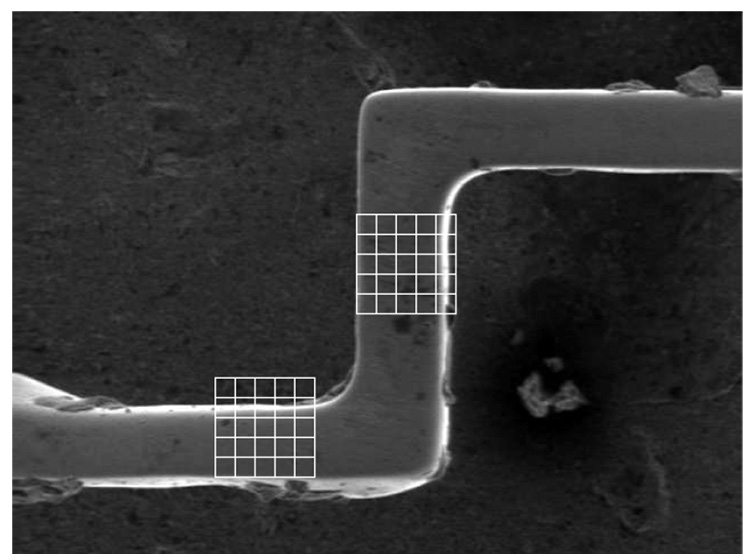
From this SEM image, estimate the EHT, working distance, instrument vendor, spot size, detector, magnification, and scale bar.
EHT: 5 kV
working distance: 4.4 mm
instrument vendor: FEI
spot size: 3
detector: SE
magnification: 0.341 K X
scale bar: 50000 nm